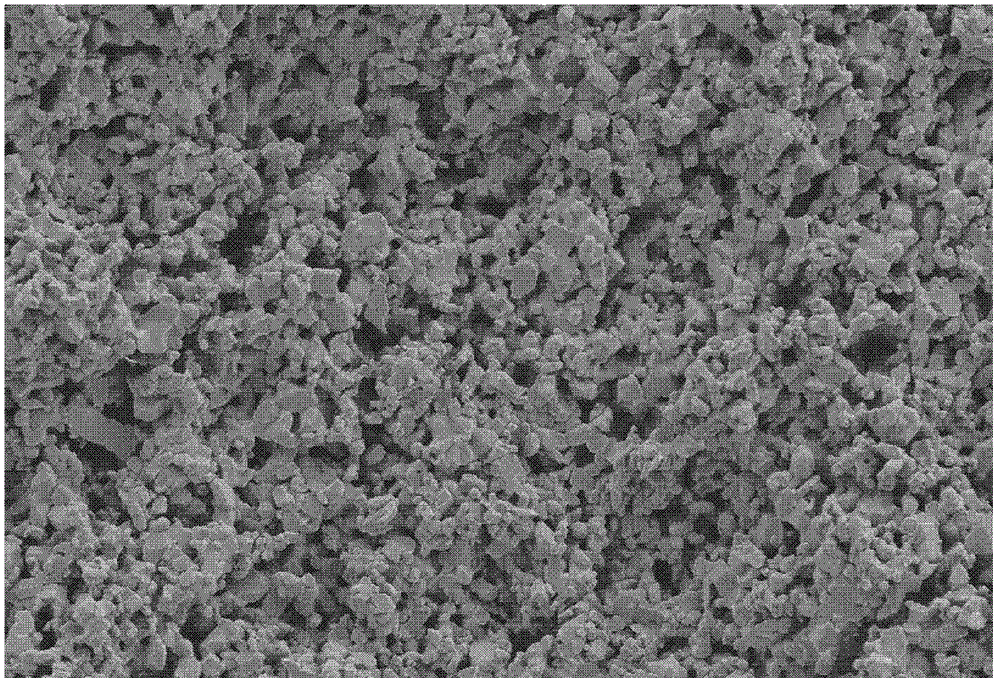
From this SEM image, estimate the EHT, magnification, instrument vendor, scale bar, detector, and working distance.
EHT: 5 kV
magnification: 5 K X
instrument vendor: Zeiss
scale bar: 10000 nm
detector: SE2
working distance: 5 mm